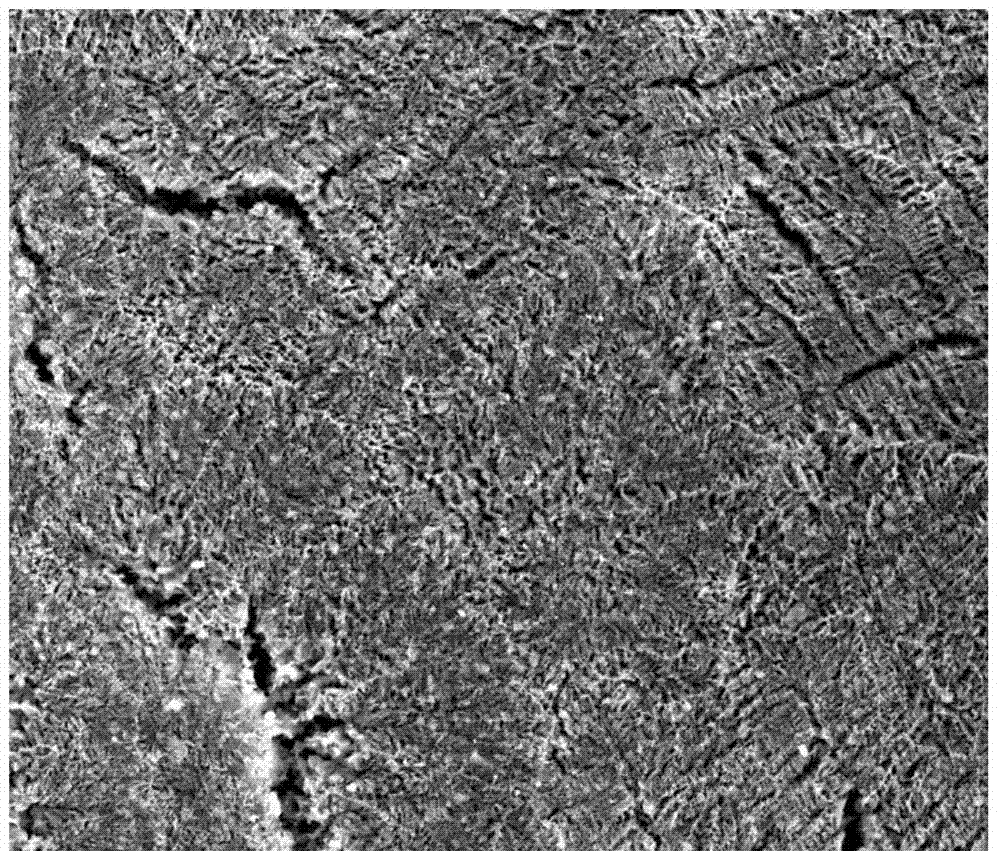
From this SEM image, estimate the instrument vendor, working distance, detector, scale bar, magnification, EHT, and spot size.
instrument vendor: FEI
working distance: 10 mm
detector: ETD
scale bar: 100000 nm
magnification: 0.6 K X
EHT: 20 kV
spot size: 3